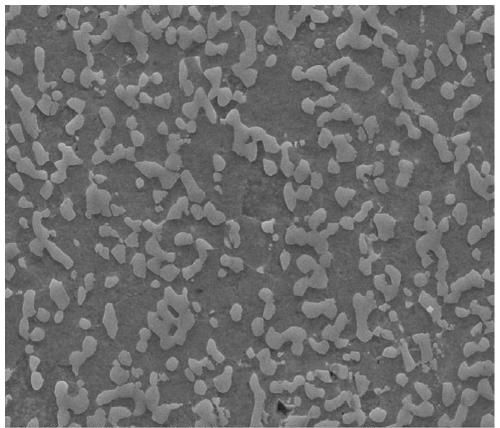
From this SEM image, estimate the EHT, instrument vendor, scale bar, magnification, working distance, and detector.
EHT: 30 kV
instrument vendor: FEI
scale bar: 20000 nm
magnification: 5 K X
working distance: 5.9 mm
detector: SE